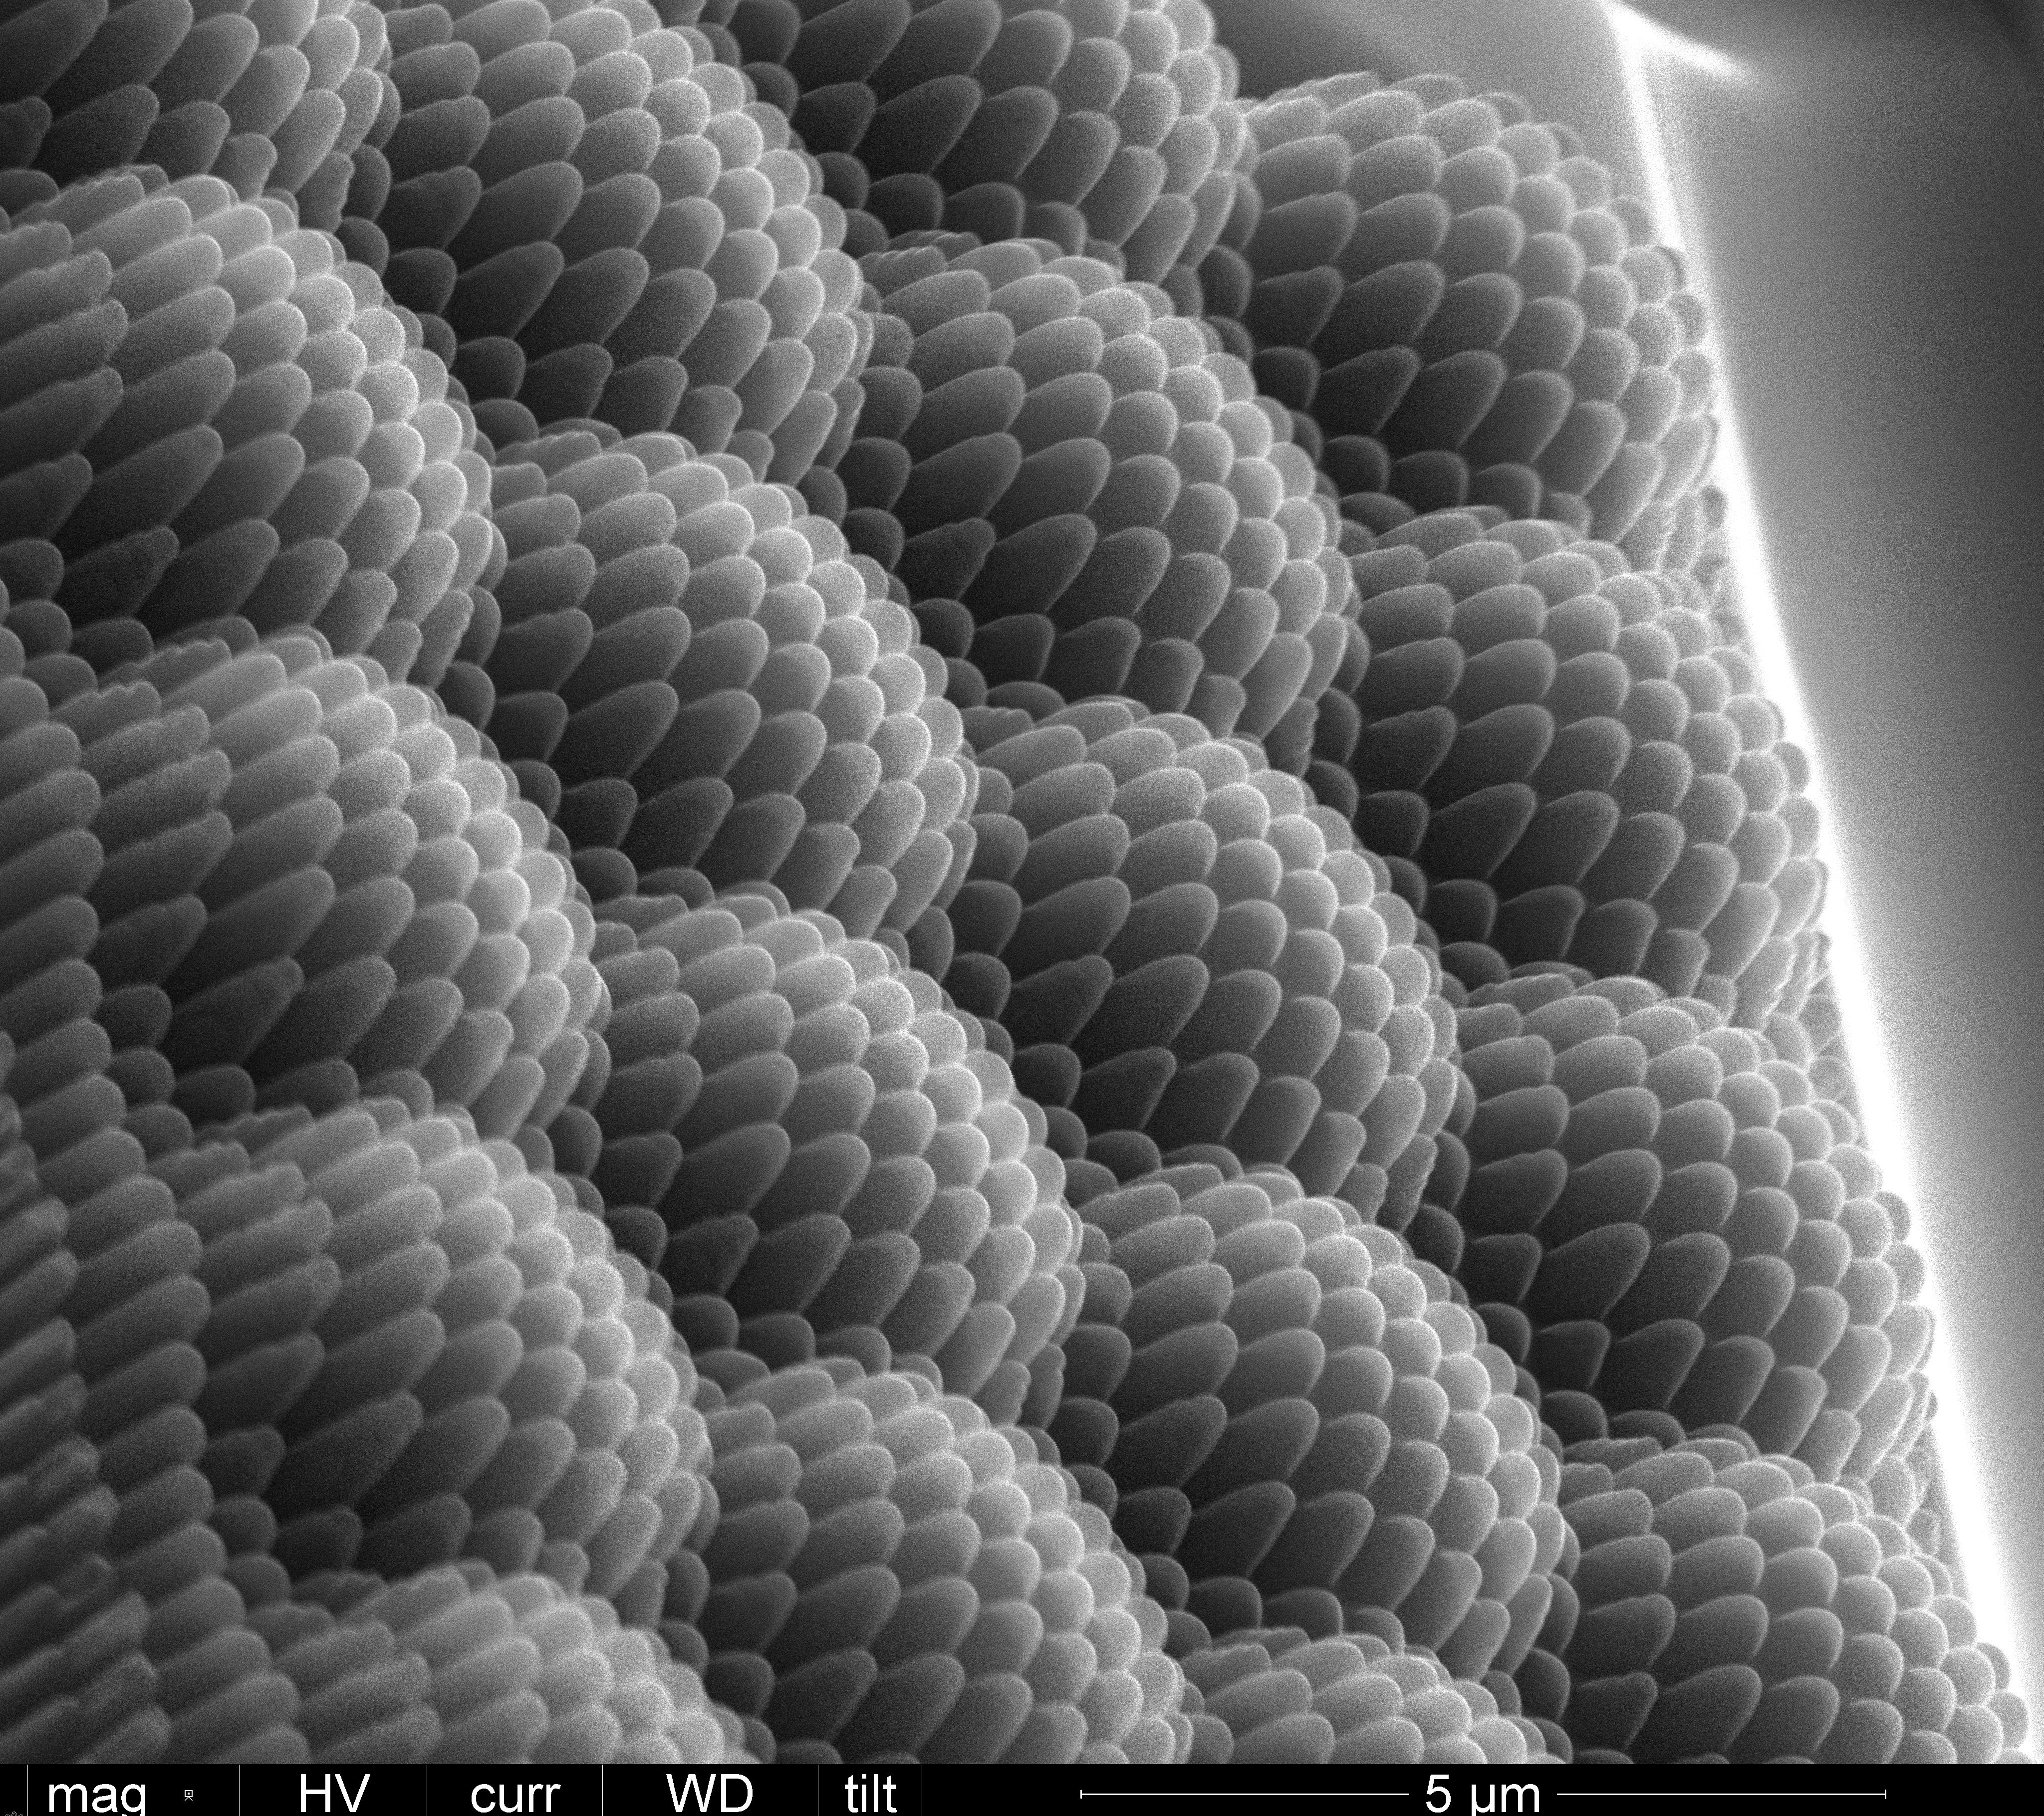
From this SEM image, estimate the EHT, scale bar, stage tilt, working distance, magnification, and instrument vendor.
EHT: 20 kV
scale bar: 5000 nm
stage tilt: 45°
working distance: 10.1 mm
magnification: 10 K X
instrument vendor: FEI